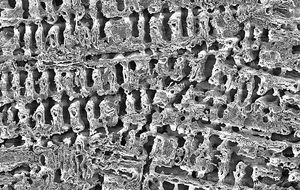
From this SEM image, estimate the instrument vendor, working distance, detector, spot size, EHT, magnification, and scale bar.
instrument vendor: FEI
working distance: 4.8 mm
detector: ETD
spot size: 3.2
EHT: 5 kV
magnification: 2 K X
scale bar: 50000 nm